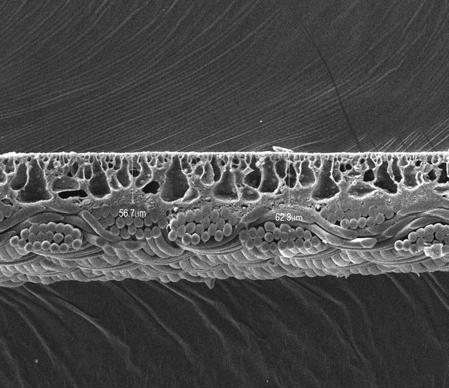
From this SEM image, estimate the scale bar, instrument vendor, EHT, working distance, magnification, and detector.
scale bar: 100000 nm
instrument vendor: JEOL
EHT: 15 kV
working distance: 8 mm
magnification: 0.2 K X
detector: LEI